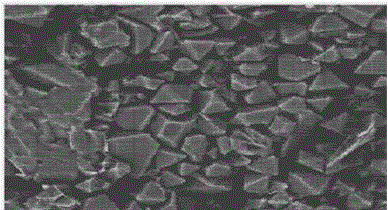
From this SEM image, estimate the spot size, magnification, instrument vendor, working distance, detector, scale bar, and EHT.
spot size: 3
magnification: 20 K X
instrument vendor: FEI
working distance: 6.1 mm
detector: TLD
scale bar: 1000 nm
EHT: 10 kV